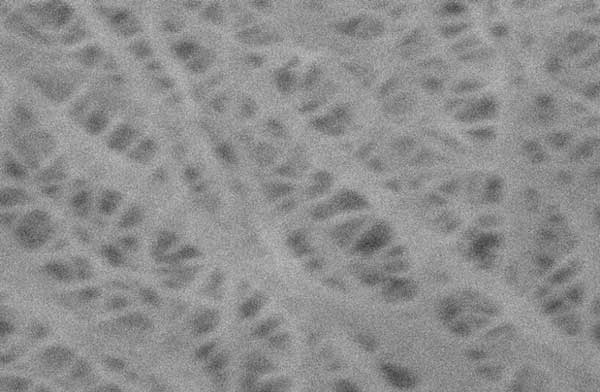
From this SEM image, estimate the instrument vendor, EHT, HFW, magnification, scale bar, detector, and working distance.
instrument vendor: FEI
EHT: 5 kV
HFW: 6.41 µm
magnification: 25 K X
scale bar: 500 nm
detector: ETD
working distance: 5.77 mm